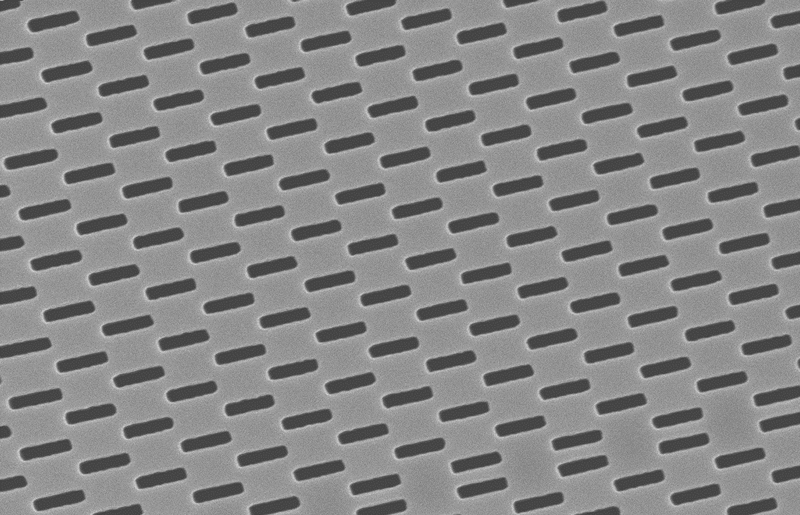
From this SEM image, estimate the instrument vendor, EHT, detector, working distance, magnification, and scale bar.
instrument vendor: Zeiss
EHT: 10 kV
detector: InLens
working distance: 1 mm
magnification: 23.89 K X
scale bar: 1000 nm